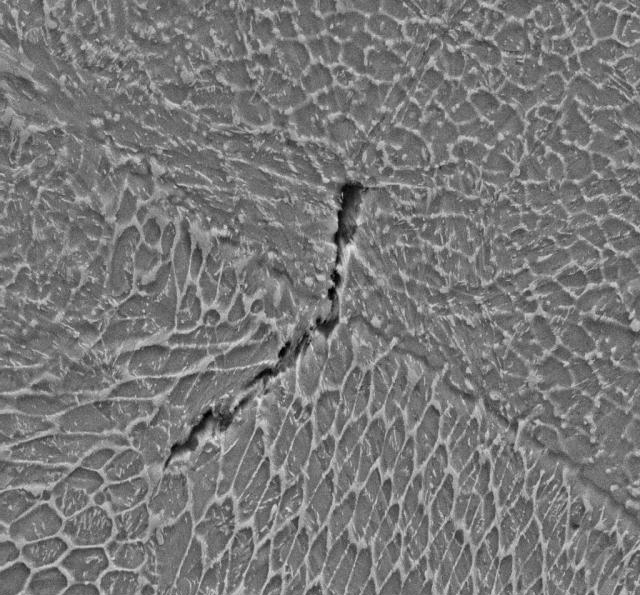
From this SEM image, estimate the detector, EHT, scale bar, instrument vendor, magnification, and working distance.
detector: SE2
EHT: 15 kV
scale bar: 3000 nm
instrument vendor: Zeiss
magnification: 10 K X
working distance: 8.3 mm